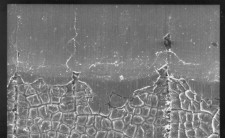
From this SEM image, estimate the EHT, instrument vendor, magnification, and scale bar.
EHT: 25 kV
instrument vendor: JEOL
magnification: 0.1 K X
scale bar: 100000 nm